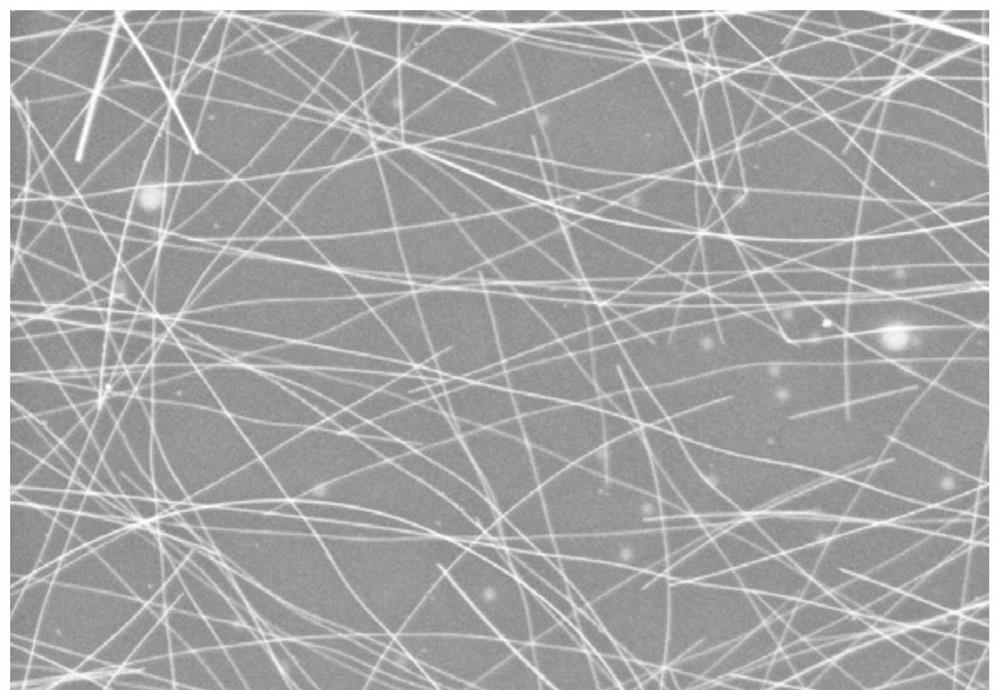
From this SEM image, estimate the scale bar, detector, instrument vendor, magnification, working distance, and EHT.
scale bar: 1000 nm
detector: InLens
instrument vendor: Zeiss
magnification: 10 K X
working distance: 4.9 mm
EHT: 5 kV